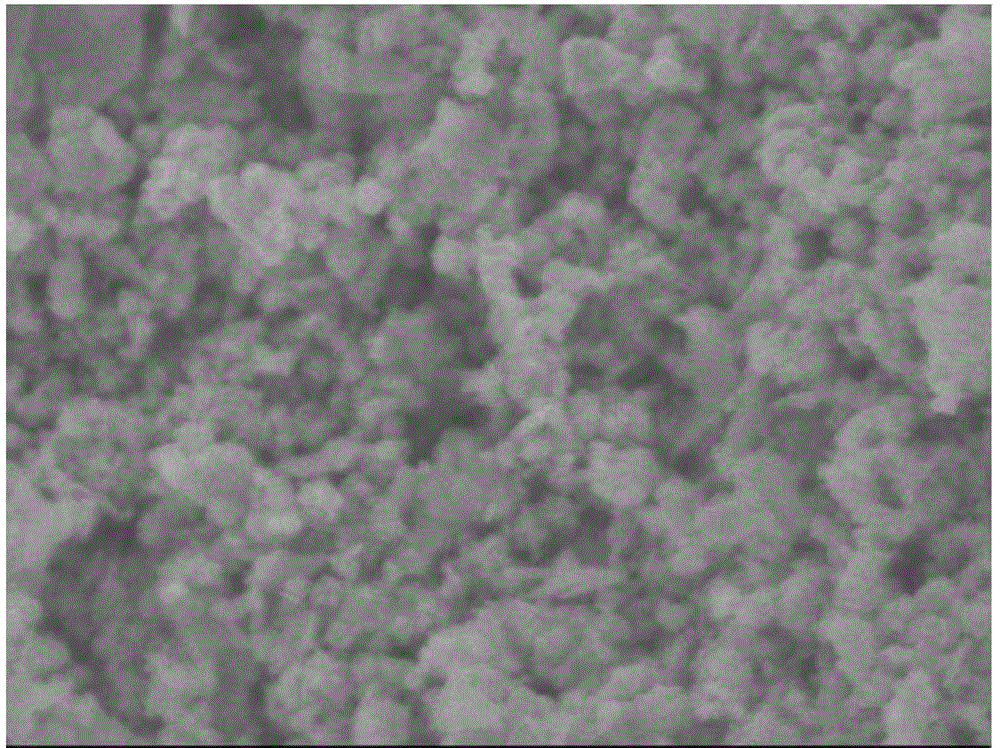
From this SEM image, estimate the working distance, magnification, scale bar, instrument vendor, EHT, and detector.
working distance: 7.9 mm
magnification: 30 K X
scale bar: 100 nm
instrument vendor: JEOL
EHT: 5 kV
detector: SEI